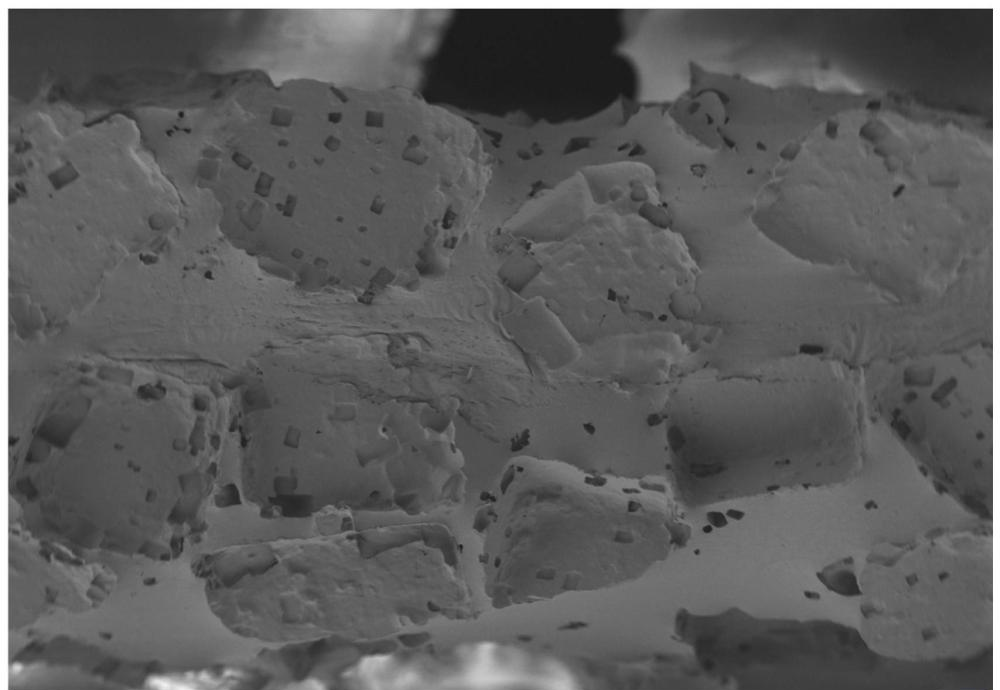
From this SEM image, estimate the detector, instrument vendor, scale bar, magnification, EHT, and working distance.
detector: SE2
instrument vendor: Zeiss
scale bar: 100000 nm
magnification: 0.07 K X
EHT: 5 kV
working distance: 13.2 mm